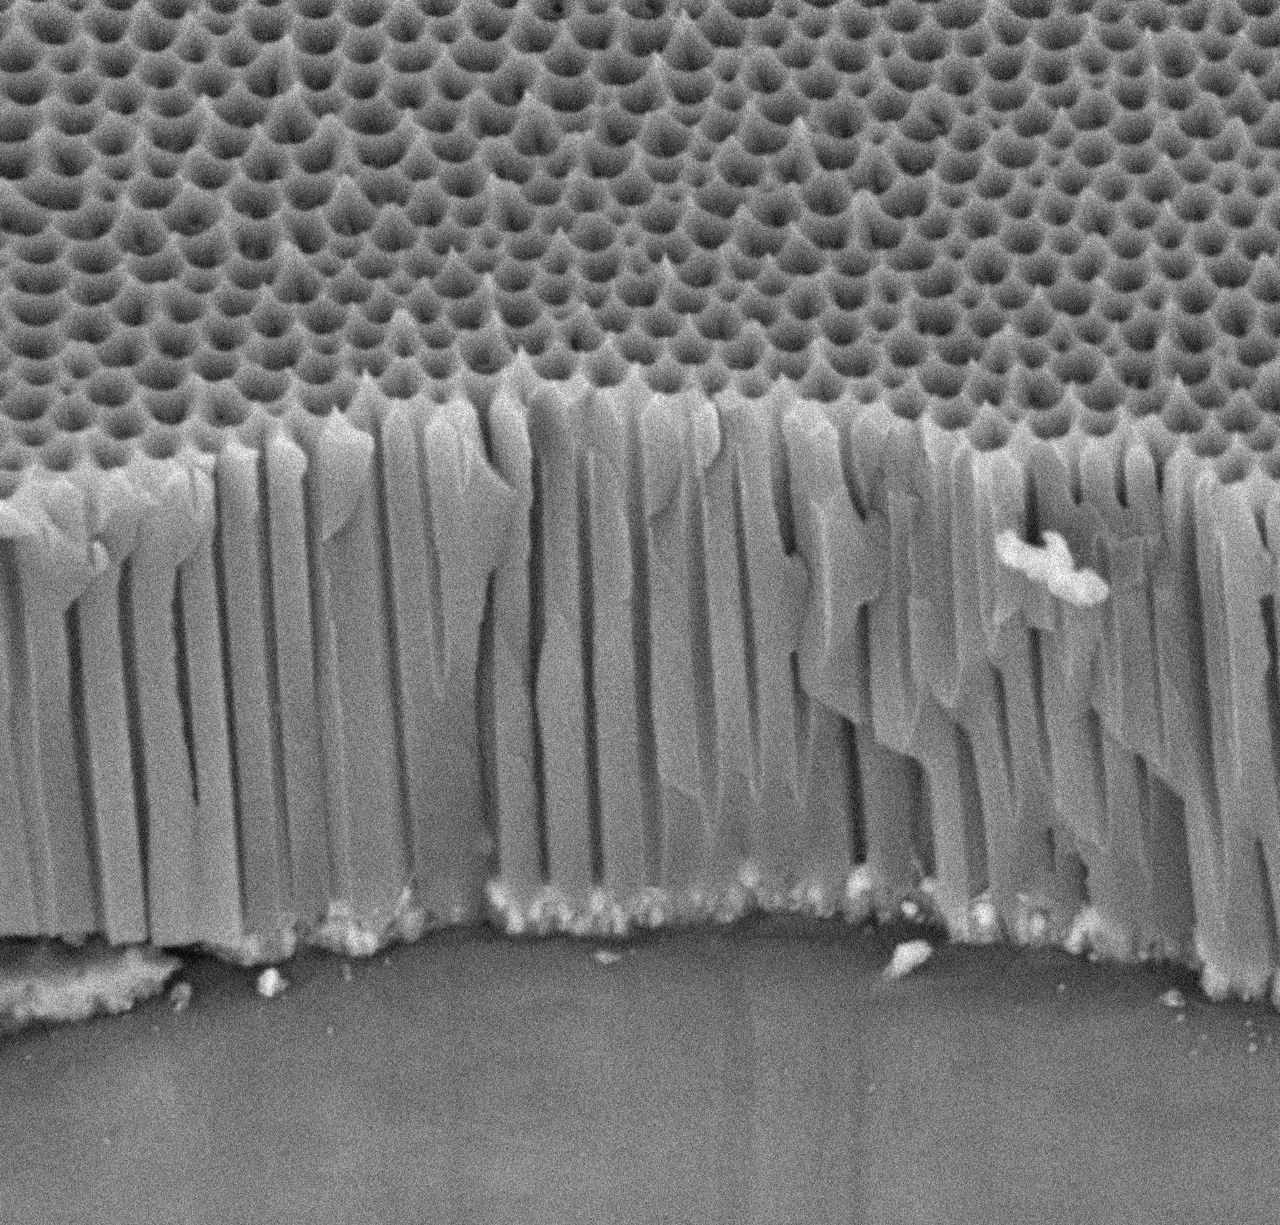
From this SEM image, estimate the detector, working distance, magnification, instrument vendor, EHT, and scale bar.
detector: BSE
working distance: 6.5 mm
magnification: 20 K X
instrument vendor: other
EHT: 15 kV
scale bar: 2000 nm